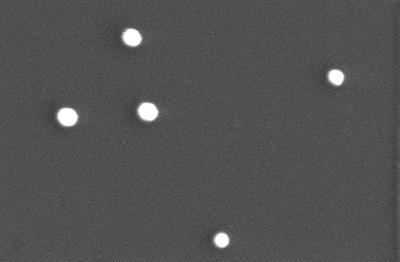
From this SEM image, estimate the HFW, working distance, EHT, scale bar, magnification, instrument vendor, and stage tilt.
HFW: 0.863 µm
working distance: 9.7 mm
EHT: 30 kV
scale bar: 200 nm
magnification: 240 K X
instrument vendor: FEI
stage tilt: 0°